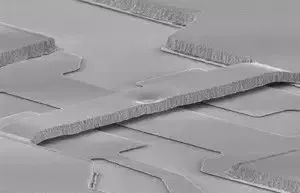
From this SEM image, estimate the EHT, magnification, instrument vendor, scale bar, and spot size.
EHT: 15 kV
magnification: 1.438 K X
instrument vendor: FEI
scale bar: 20000 nm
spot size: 3